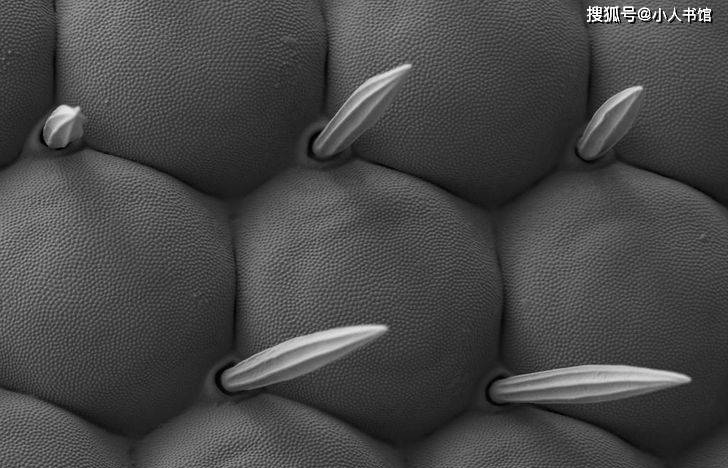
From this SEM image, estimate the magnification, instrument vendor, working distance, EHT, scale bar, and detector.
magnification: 3 K X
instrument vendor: Zeiss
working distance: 4.2 mm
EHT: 1 kV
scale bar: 2000 nm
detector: SE2